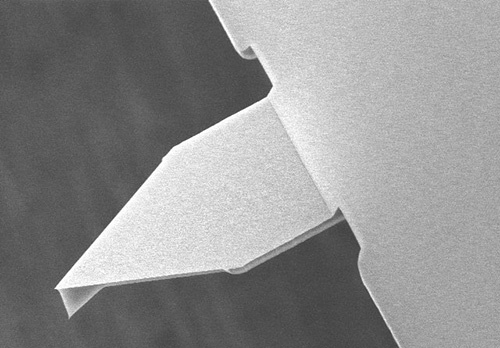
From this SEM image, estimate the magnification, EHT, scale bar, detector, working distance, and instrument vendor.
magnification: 1.3 K X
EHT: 15 kV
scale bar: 40000 nm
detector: SE(U)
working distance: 7.5 mm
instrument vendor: Hitachi